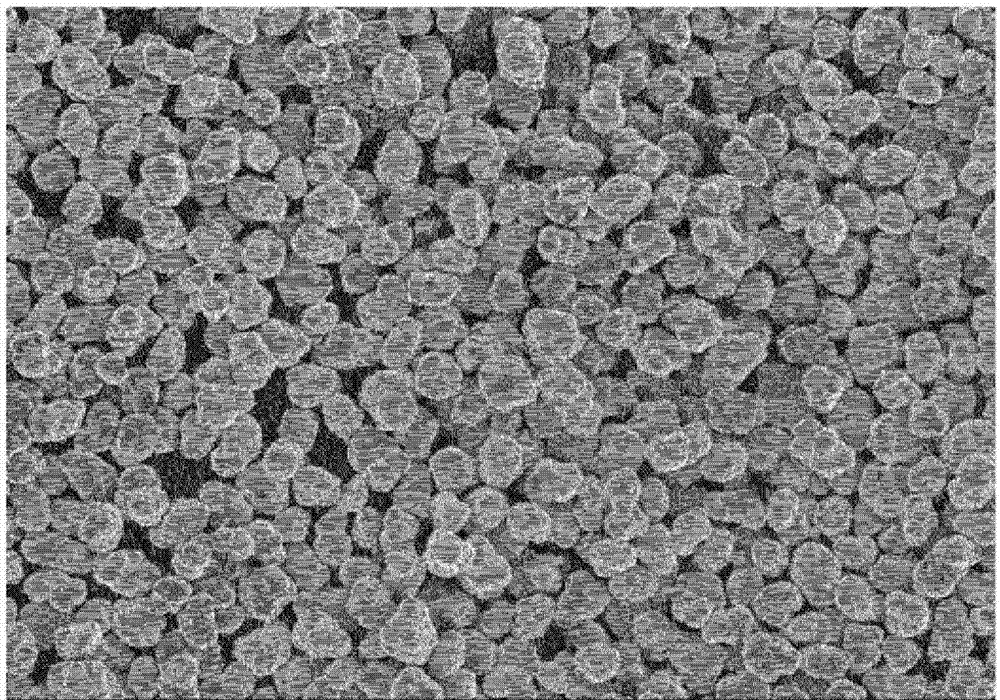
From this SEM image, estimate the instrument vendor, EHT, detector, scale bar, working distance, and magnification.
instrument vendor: Hitachi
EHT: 5 kV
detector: SE(M)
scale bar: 50000 nm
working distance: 12.2 mm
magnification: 1 K X